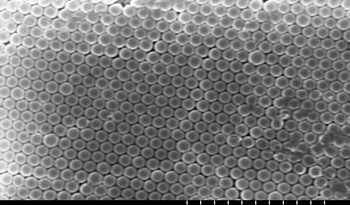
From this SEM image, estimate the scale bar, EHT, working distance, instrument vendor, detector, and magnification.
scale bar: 5000 nm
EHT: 15 kV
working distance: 12.3 mm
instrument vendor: Hitachi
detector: SE(M)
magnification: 10 K X